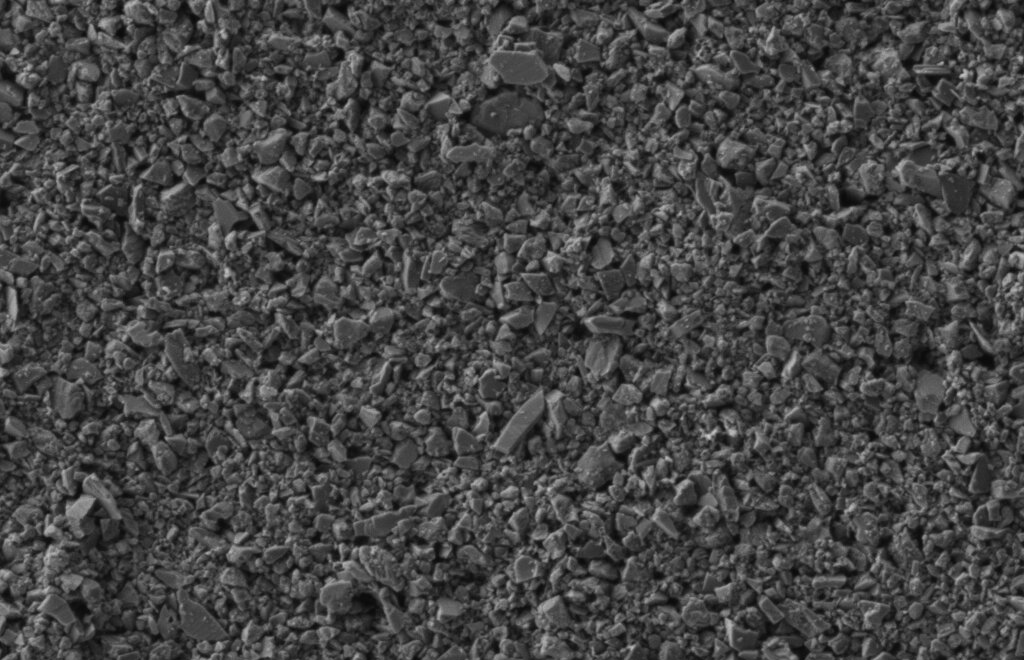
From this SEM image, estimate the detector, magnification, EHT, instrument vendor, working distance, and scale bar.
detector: SE2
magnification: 20 K X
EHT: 1 kV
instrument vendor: Zeiss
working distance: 4.3 mm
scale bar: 200 nm